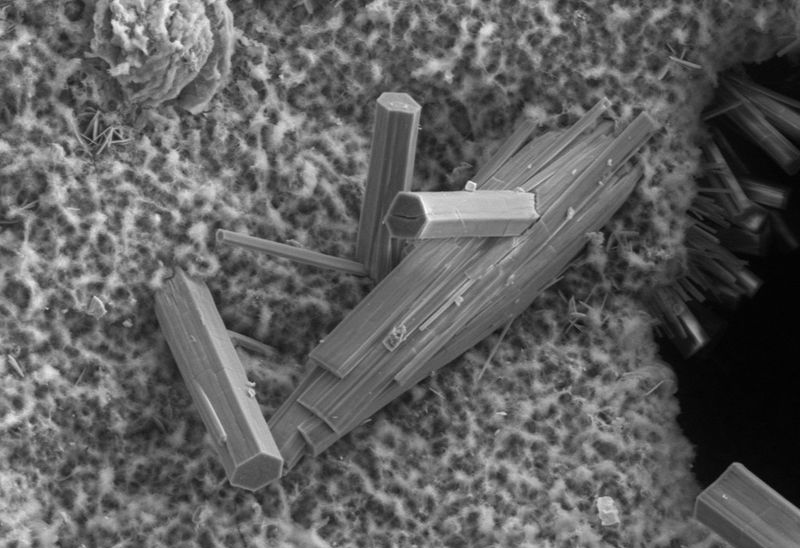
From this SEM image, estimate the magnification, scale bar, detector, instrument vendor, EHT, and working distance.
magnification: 0.266 K X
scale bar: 20000 nm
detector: VPSE G3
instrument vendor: Zeiss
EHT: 15 kV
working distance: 9.5 mm